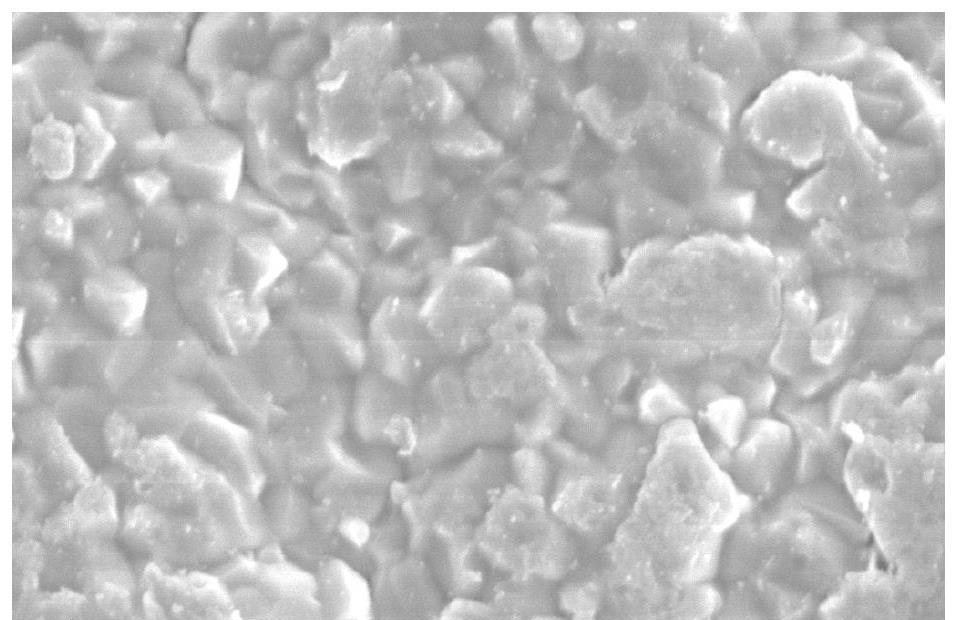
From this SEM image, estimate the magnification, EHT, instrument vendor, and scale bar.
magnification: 4 K X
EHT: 21 kV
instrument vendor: other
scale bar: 10000 nm